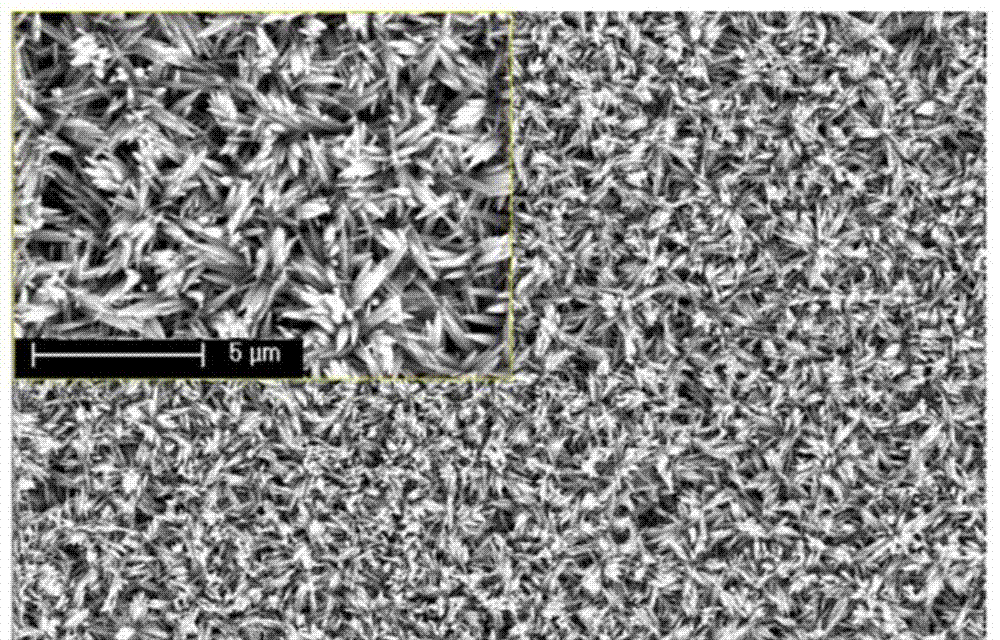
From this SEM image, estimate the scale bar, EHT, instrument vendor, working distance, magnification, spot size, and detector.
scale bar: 10000 nm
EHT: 20 kV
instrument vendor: FEI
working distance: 5.4 mm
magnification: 5 K X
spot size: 3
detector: TLD